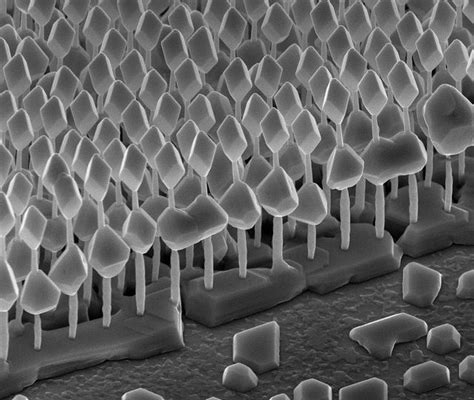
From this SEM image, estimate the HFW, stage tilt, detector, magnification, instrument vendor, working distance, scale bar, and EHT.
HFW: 6 µm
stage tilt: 50°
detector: SE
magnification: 50 K X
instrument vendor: FEI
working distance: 5.1 mm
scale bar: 1000 nm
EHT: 7.8 kV